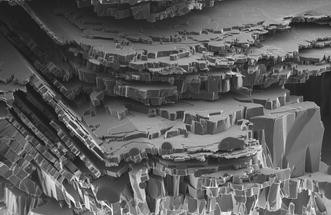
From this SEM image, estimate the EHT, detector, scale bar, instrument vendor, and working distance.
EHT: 1 kV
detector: InLens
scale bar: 1000 nm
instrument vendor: Zeiss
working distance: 2 mm